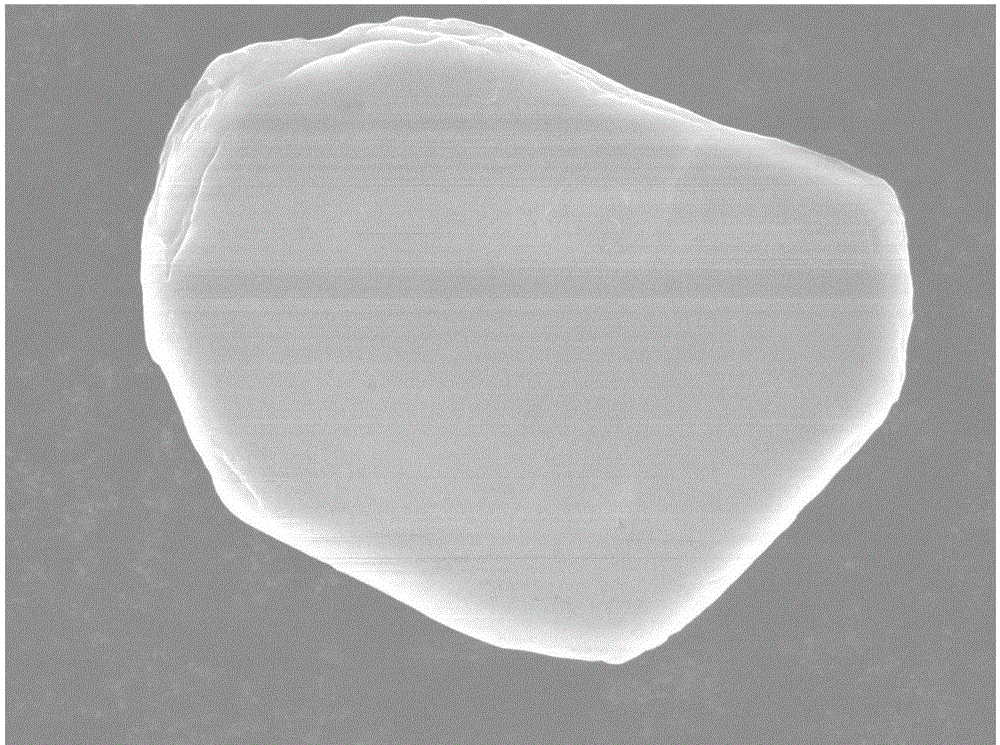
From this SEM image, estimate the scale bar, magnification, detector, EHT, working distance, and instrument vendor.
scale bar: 1000 nm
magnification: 3.3 K X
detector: SEI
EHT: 15 kV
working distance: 7.6 mm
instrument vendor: JEOL